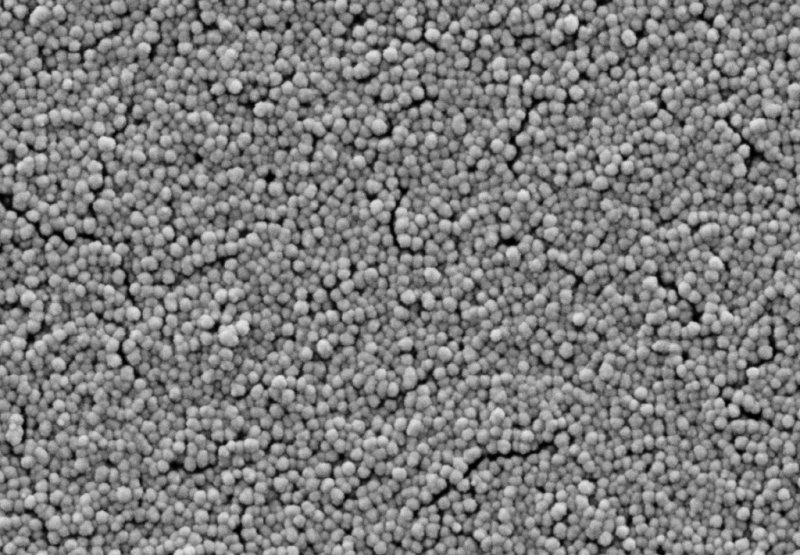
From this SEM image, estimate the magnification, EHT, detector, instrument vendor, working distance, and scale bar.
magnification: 50 K X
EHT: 3 kV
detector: SE2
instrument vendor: Zeiss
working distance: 7.2 mm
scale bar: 200 nm